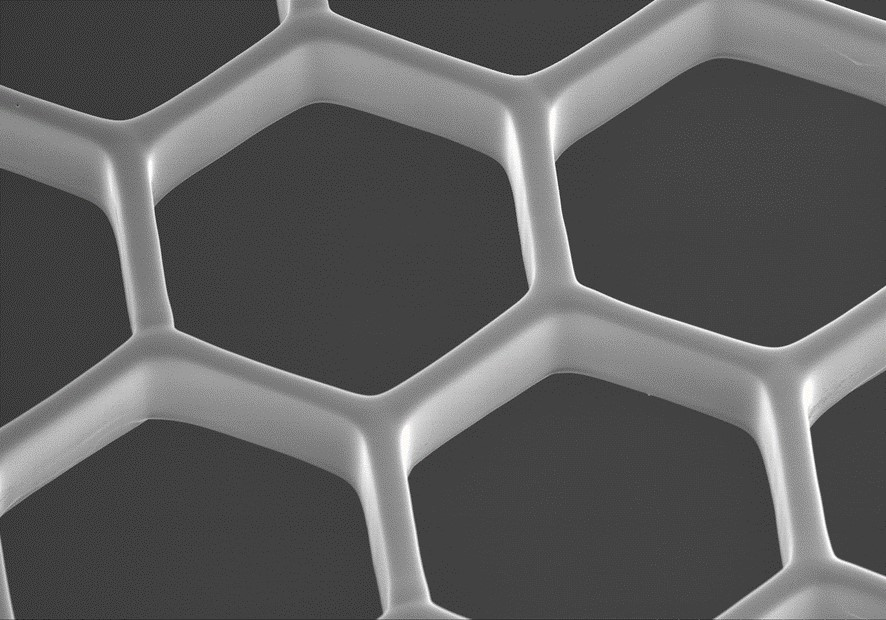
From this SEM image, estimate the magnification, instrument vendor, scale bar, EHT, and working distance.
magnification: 0.3 K X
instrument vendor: Hitachi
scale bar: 100000 nm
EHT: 15 kV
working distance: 13.3 mm